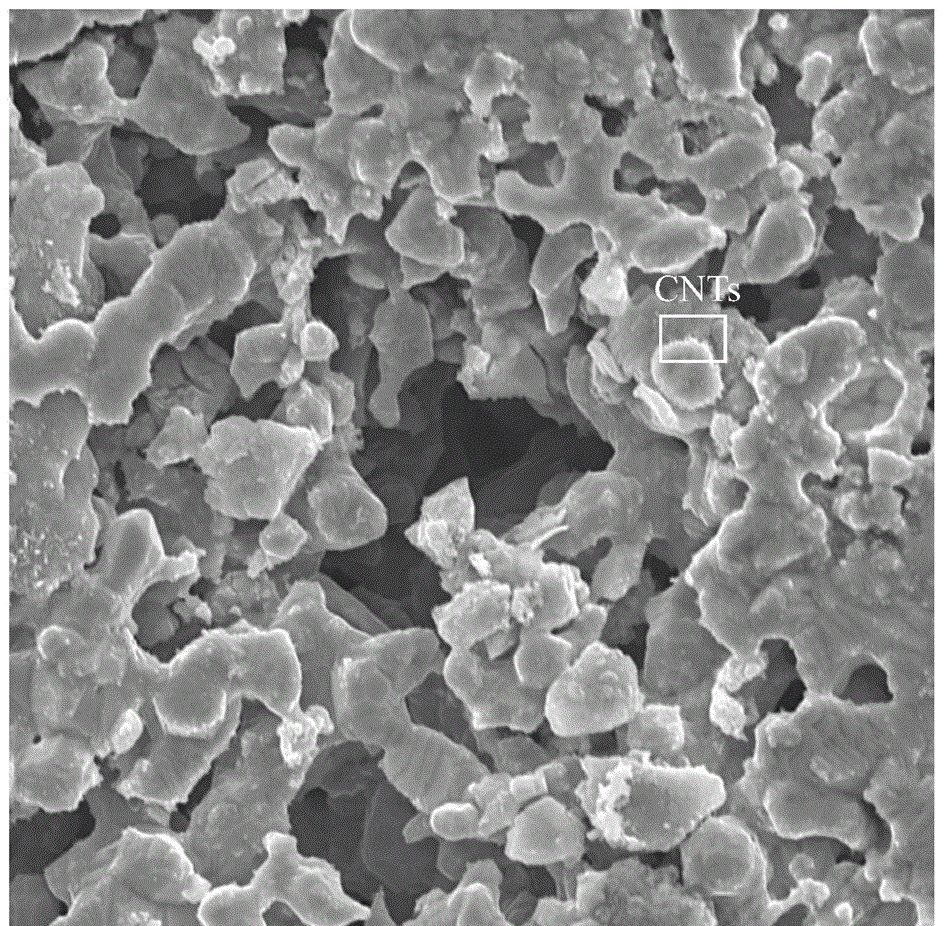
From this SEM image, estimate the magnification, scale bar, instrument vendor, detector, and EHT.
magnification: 10 K X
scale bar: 8000 nm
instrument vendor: Thermo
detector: SED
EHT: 15 kV